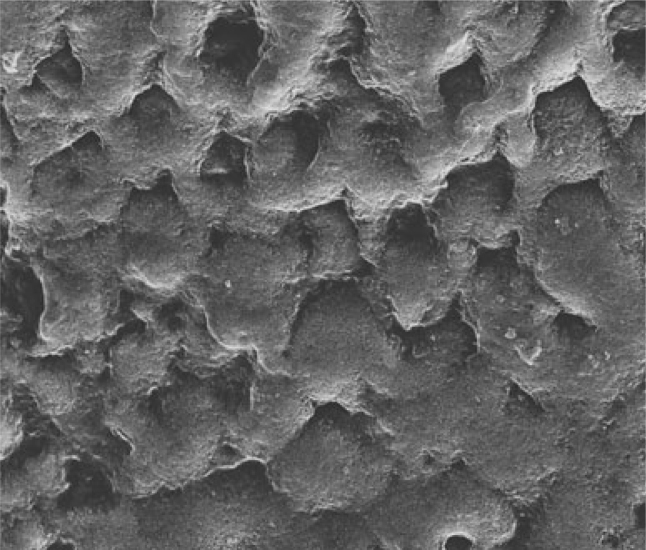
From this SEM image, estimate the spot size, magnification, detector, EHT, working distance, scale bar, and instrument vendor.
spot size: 2.5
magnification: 6 K X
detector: ETD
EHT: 20 kV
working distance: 11 mm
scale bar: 20000 nm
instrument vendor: FEI